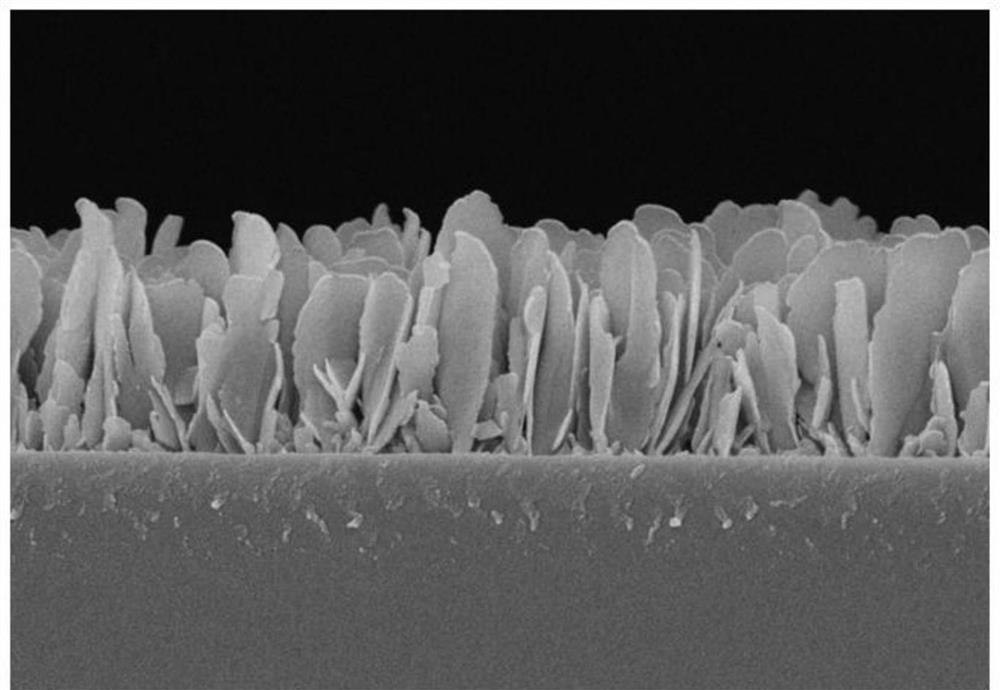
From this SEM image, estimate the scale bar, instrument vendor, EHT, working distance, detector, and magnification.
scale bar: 200 nm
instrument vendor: Zeiss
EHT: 5 kV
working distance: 2.7 mm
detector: InLens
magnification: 20 K X